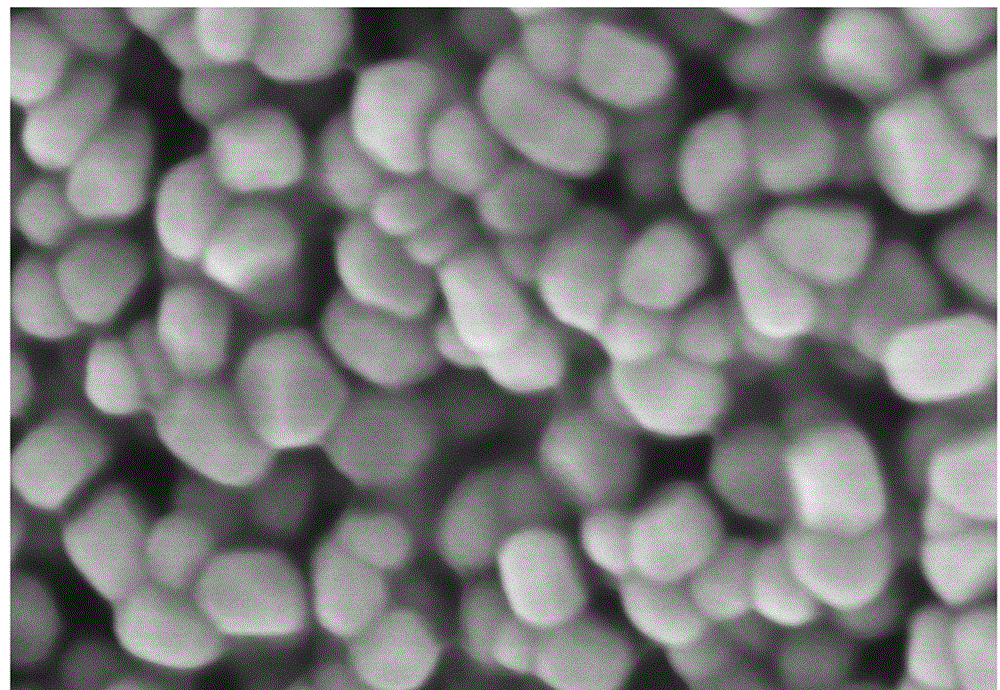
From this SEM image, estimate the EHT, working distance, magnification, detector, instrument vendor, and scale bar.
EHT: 3 kV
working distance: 2.4 mm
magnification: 200 K X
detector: SE(U)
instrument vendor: Hitachi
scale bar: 200 nm